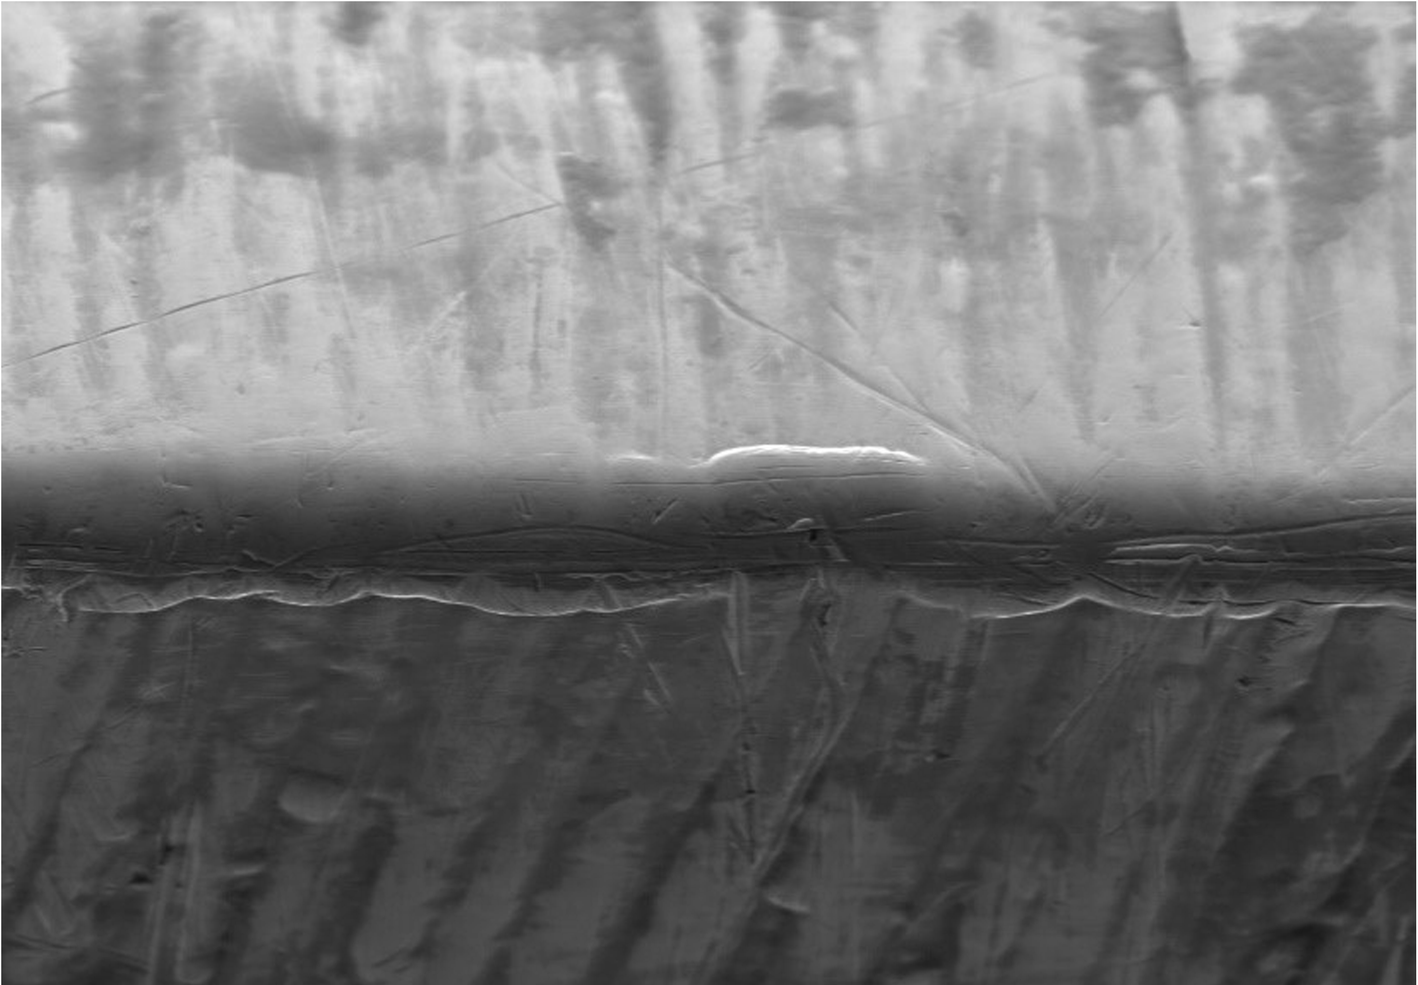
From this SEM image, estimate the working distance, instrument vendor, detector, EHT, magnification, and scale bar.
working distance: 15.4 mm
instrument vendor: Hitachi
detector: SE(M)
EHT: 15 kV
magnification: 0.5 K X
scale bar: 100000 nm